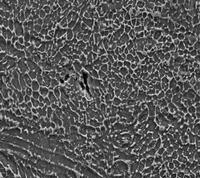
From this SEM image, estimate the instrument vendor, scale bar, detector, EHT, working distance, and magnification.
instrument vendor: Zeiss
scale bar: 2000 nm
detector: SE2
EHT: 15 kV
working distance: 7.9 mm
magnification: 10 K X